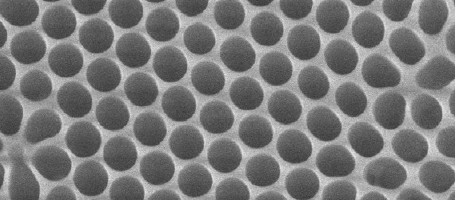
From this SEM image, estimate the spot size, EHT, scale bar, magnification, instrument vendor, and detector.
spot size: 3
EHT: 30 kV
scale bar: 400 nm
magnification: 200 K X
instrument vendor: FEI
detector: SE